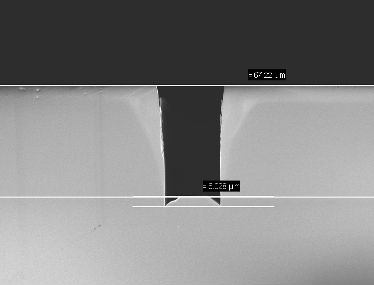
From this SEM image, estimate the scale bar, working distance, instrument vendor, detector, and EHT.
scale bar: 20000 nm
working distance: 3 mm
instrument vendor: Zeiss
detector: InLens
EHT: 5 kV